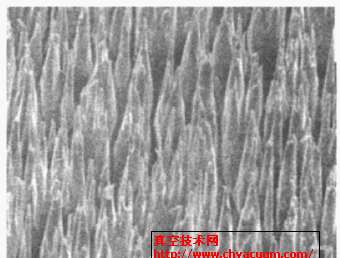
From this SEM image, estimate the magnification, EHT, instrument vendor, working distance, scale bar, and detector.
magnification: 10 K X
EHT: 5 kV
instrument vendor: JEOL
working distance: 5.5 mm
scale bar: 1000 nm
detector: SEI